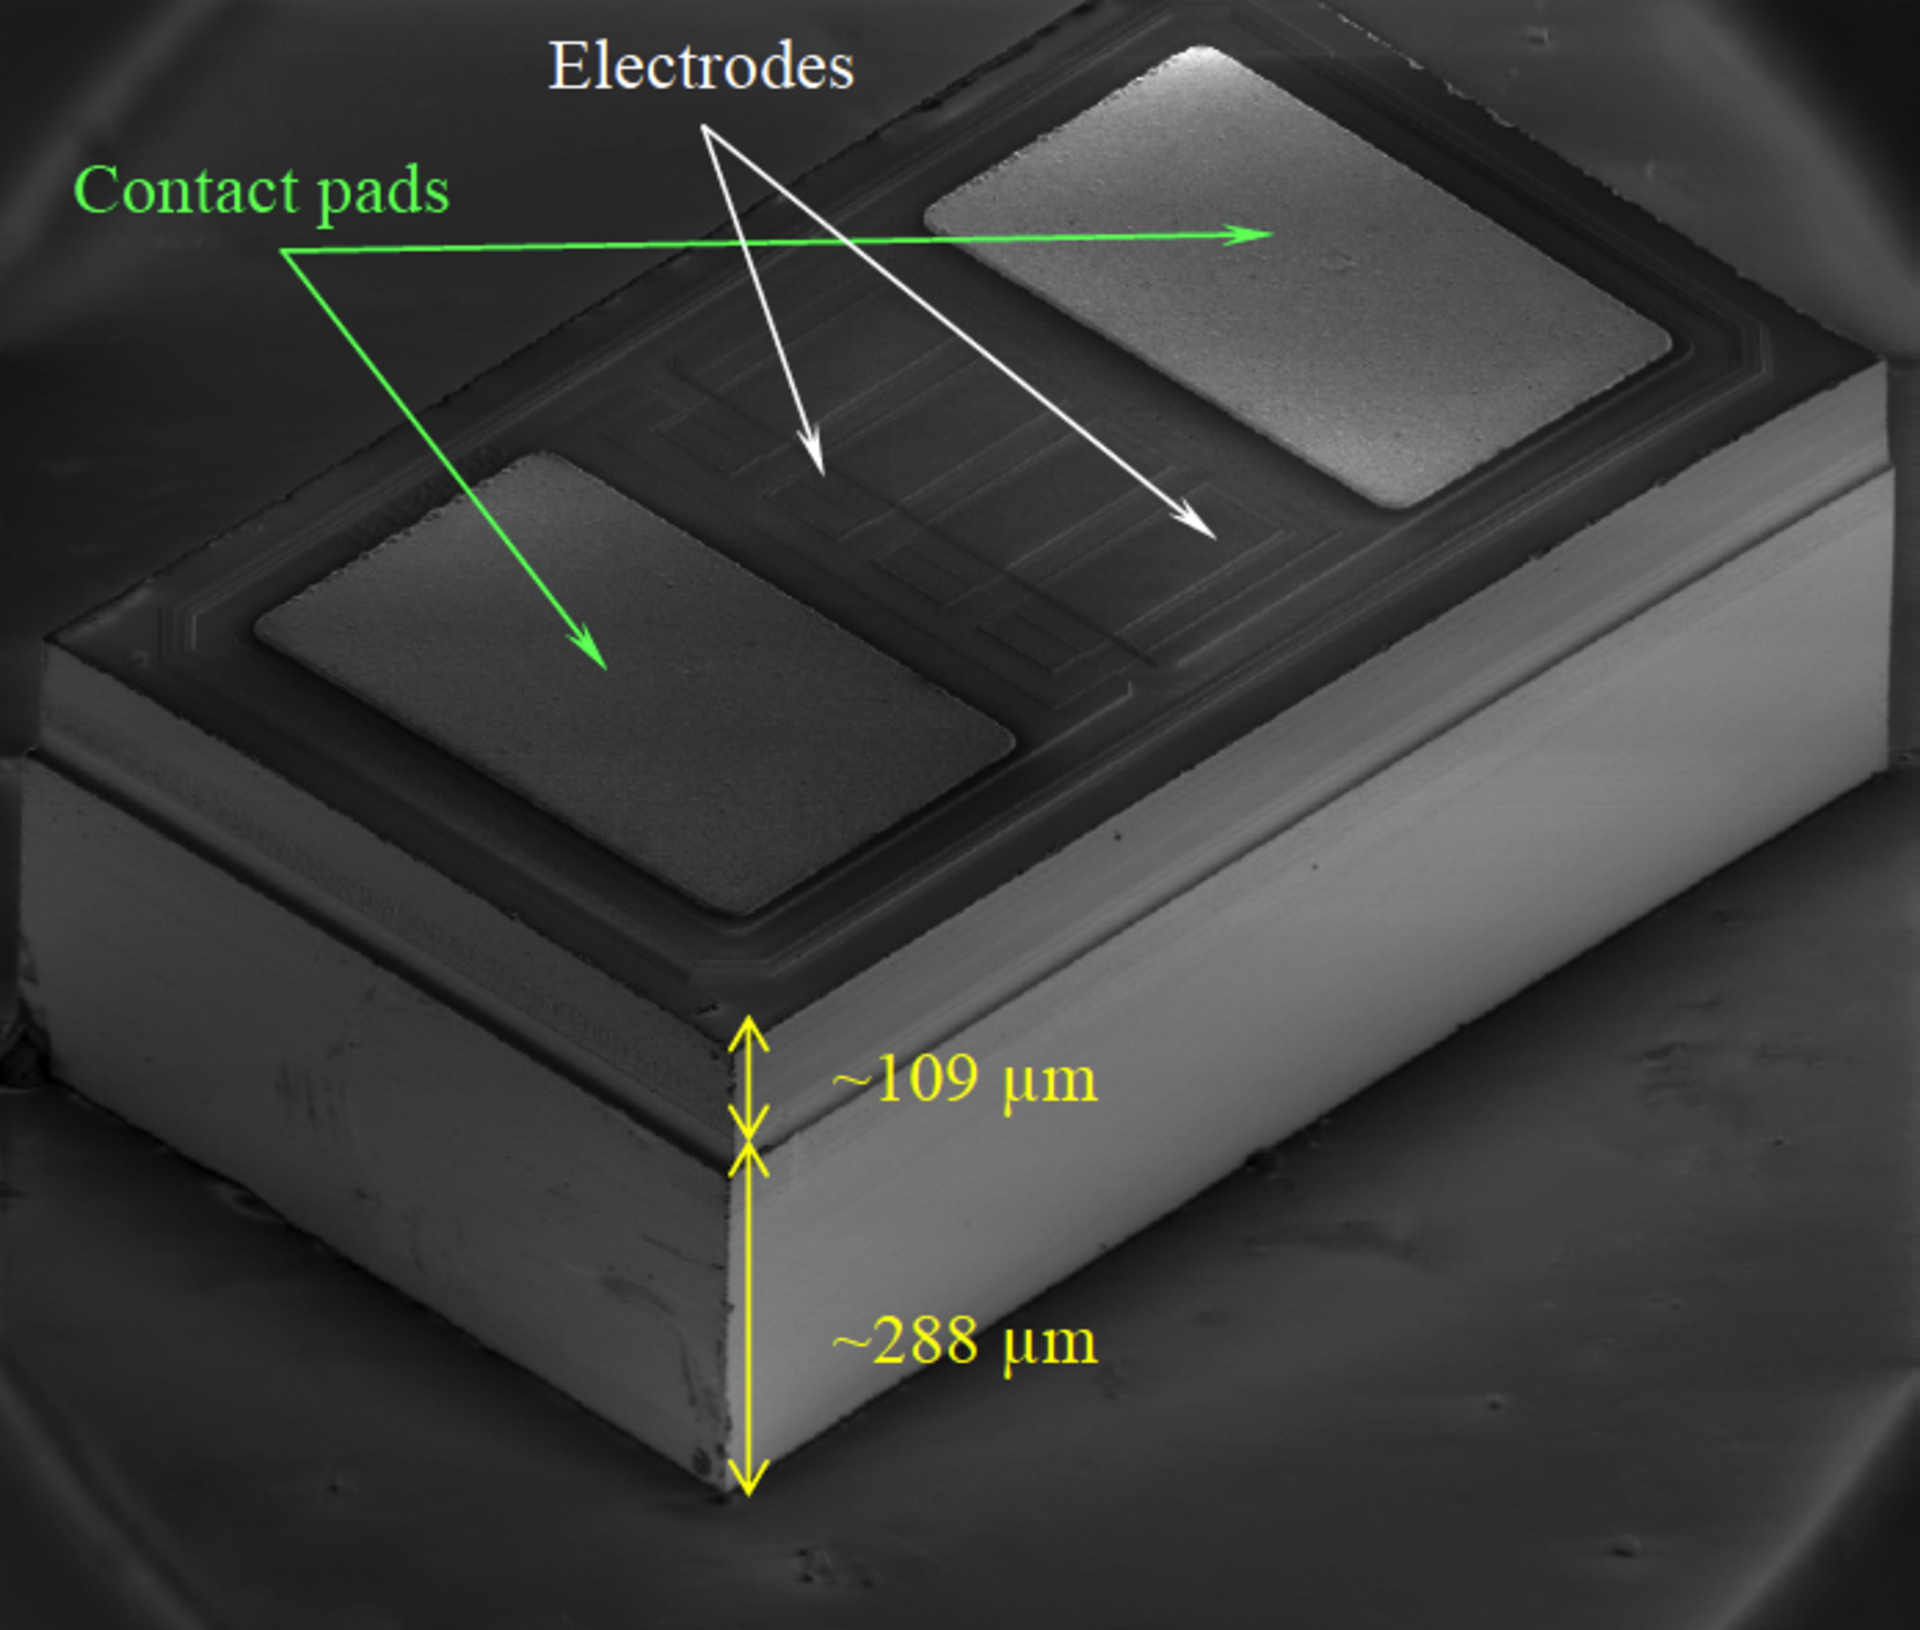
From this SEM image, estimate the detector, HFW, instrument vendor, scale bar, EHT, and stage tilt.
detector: ETD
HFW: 1400 µm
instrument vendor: FEI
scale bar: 400000 nm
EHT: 2 kV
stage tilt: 52°